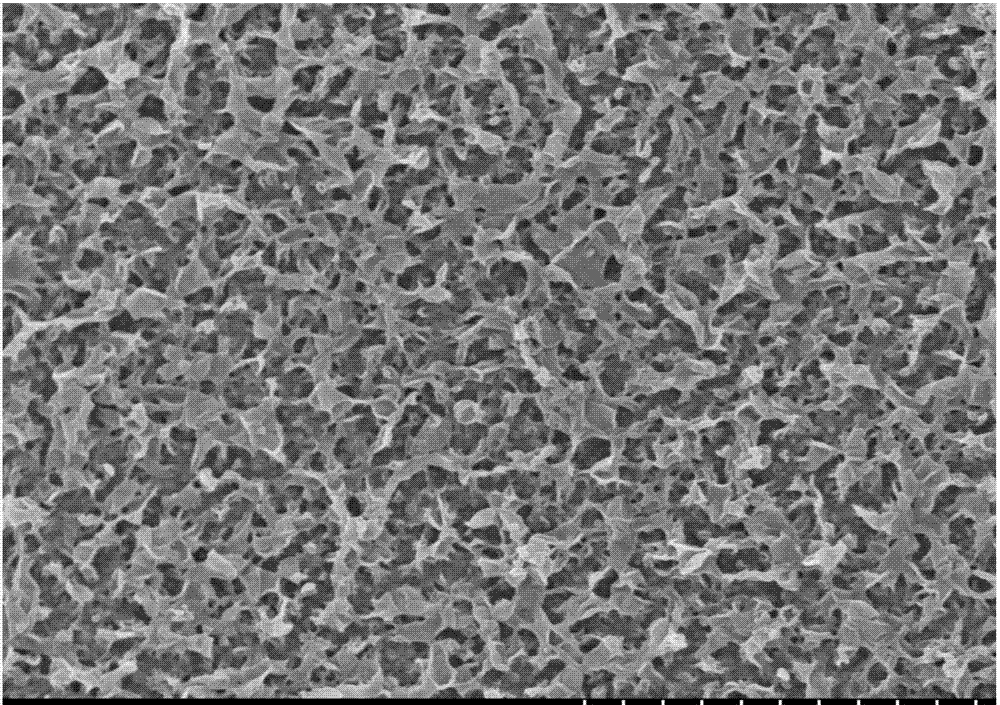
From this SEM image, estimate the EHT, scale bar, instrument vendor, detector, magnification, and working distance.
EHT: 5 kV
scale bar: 5000 nm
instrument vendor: Hitachi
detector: SE(M,LA0)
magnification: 10 K X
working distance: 3.7 mm